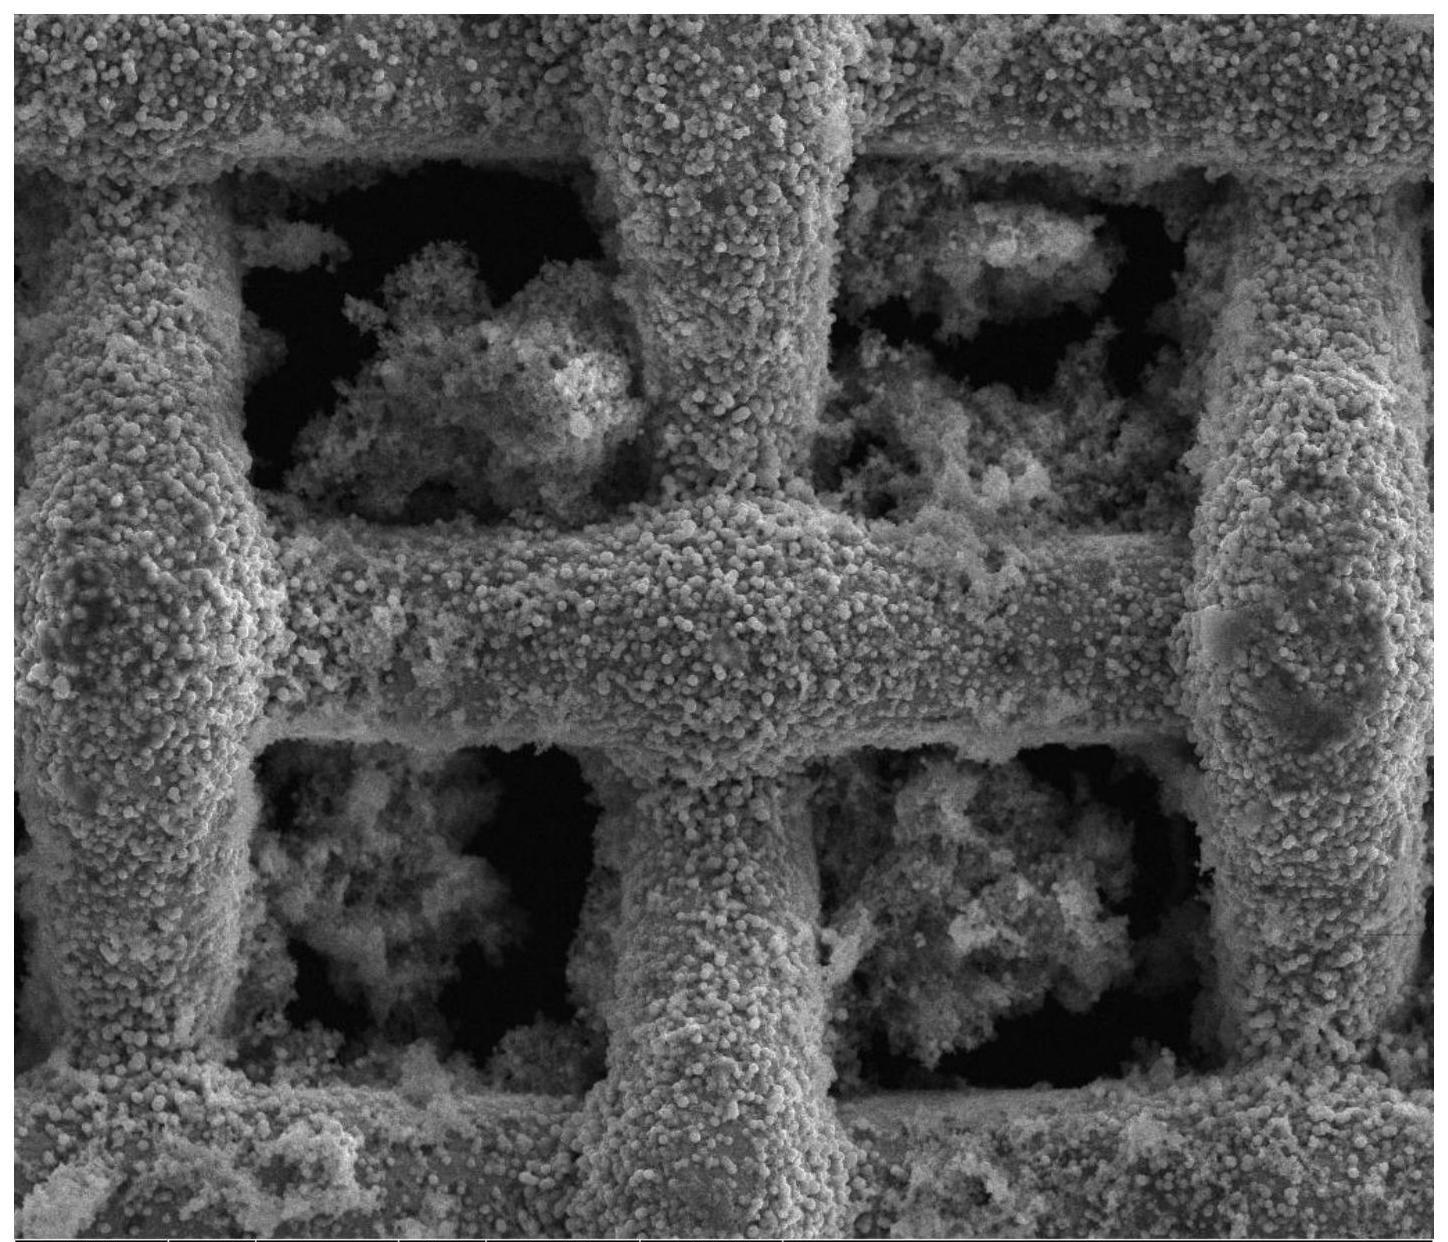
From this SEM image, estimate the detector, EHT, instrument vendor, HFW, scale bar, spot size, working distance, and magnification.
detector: ETD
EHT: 25 kV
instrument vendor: FEI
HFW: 249 µm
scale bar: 100000 nm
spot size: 3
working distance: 14.8 mm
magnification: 1.2 K X